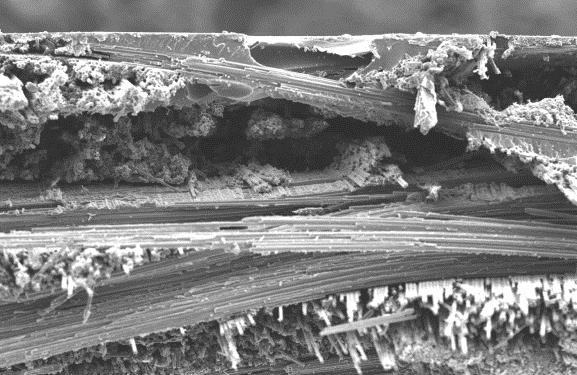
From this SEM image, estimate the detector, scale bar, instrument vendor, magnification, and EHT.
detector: SEI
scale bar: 100000 nm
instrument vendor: JEOL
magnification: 0.1 K X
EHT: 15 kV